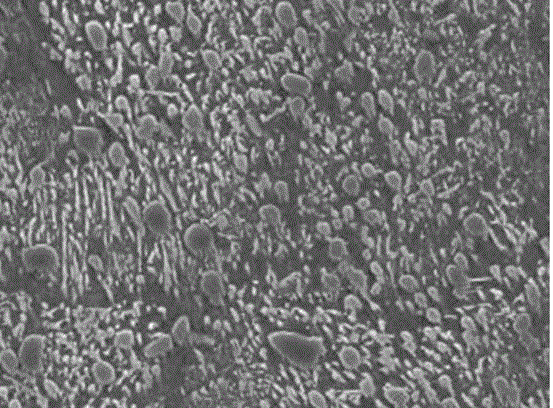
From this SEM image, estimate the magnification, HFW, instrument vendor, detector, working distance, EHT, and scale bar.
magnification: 5 K X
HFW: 29.8 µm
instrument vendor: FEI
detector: ETD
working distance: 10.2 mm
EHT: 20 kV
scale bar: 10000 nm